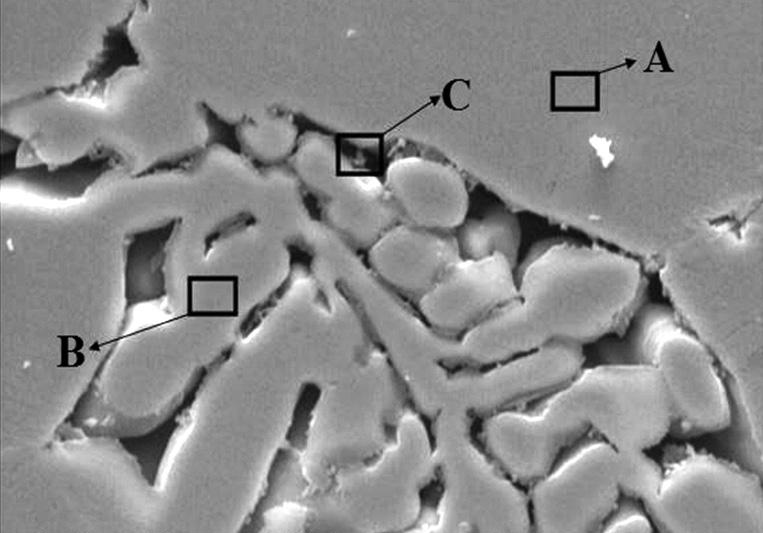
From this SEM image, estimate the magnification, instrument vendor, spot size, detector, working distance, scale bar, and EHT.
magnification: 2 K X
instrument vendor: FEI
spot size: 4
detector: SE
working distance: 12.1 mm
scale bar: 10000 nm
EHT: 25 kV